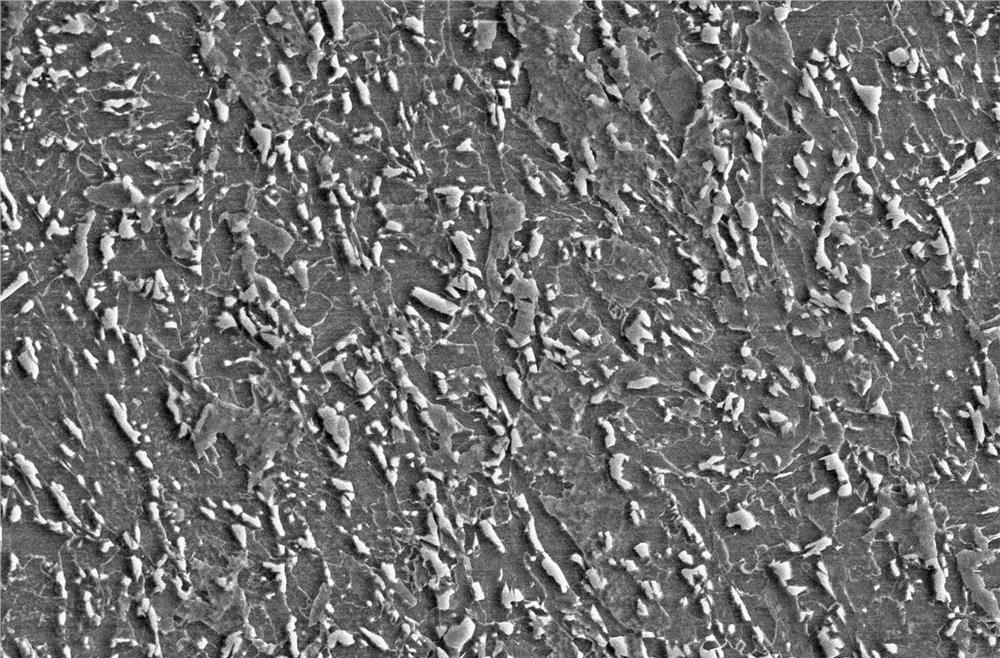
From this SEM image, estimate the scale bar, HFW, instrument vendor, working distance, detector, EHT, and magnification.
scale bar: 50000 nm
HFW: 118 µm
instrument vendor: FEI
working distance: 6.8 mm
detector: ETD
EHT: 30 kV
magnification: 3.5 K X